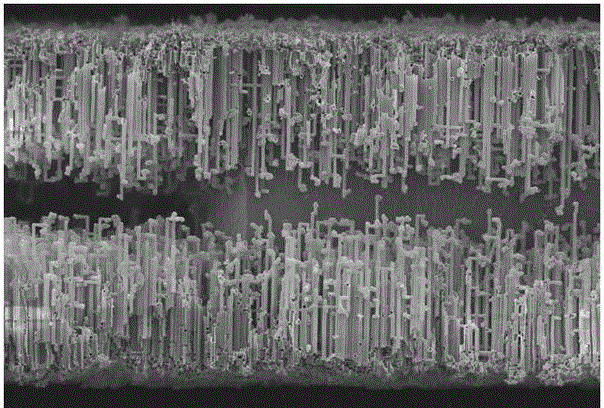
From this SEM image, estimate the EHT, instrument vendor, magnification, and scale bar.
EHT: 10 kV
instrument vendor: other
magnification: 0.6 K X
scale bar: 100000 nm